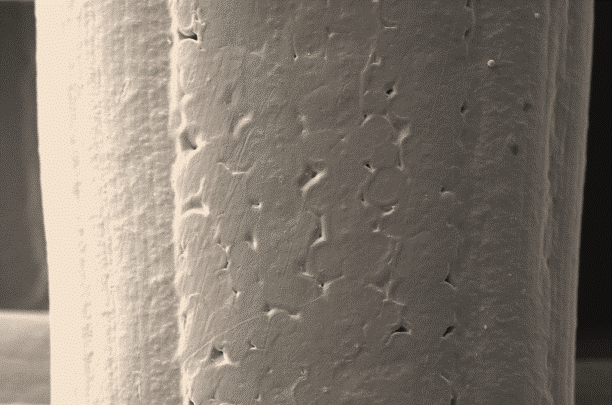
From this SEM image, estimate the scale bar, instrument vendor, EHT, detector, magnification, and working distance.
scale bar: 30000 nm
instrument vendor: Zeiss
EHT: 10 kV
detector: SE1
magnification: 0.5 K X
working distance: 13.5 mm